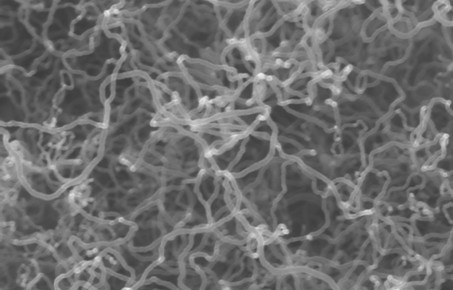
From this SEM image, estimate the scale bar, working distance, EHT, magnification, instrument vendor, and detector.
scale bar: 200 nm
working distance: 6.9 mm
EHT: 8 kV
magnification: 100 K X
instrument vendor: Zeiss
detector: InLens